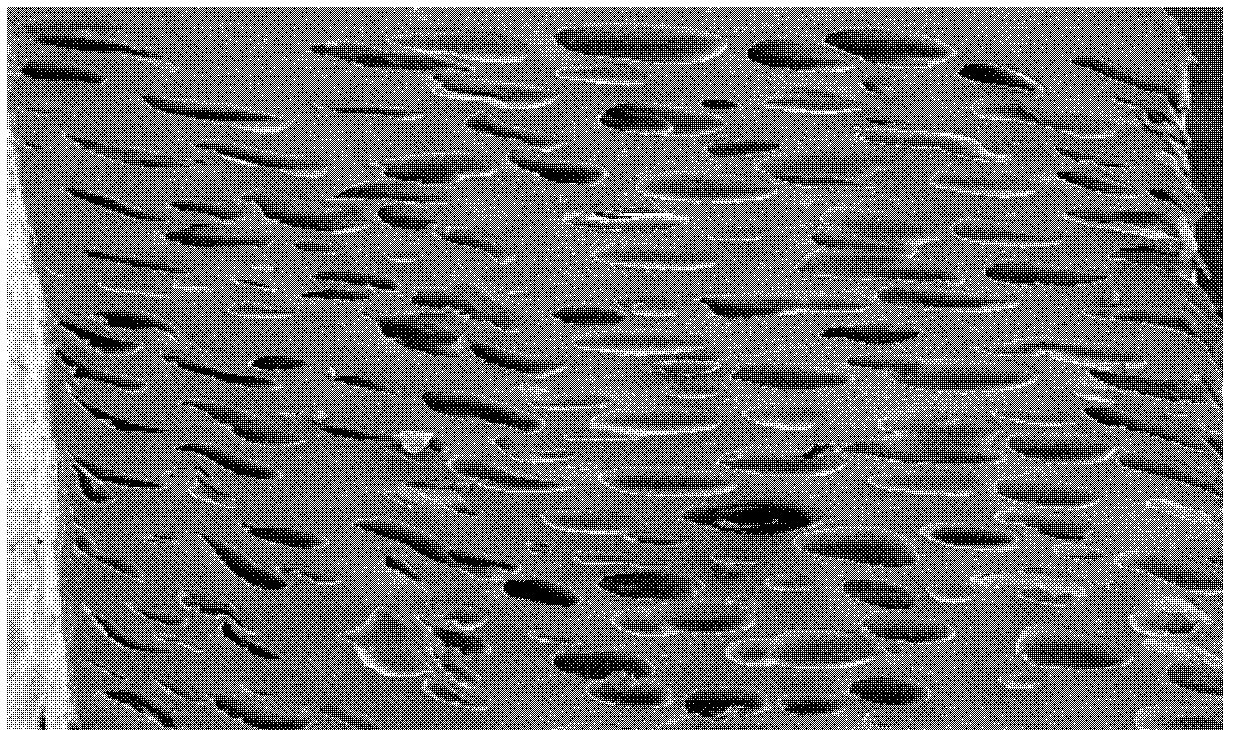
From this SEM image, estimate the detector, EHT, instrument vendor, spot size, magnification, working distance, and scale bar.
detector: SE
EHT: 5 kV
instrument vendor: FEI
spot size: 3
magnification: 0.04 K X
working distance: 8 mm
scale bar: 500000 nm